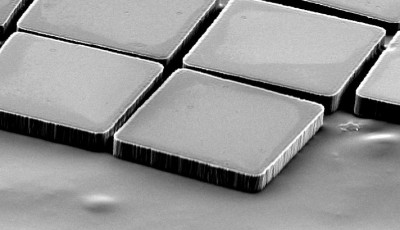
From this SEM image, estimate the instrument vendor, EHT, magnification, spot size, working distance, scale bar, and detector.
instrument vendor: FEI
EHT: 12 kV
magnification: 0.5 K X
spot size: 5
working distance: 16.8 mm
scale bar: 50000 nm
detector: SE